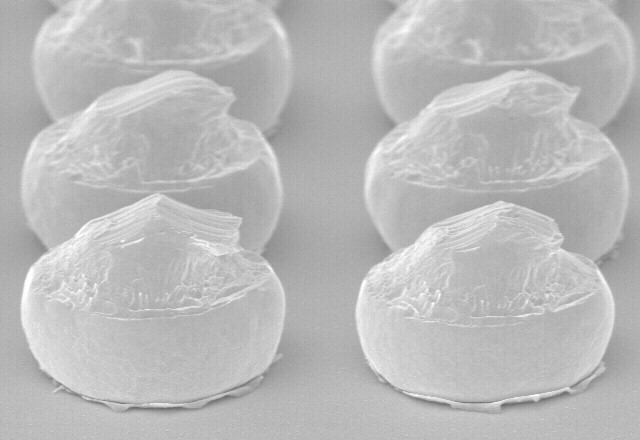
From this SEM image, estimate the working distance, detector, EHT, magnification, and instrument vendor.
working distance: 29.5 mm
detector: SE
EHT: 20 kV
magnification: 0.6 K X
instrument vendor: other